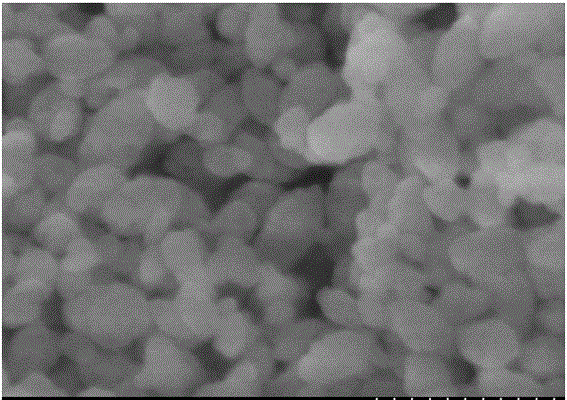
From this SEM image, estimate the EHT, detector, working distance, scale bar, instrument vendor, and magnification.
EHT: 5 kV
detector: SE(M)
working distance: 9.2 mm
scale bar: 200 nm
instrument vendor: Hitachi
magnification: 200 K X